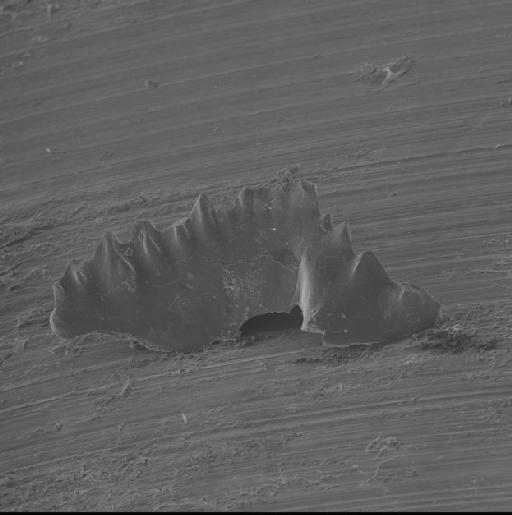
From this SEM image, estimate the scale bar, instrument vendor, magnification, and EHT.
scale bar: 300000 nm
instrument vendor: JEOL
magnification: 0.1 K X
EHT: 15 kV